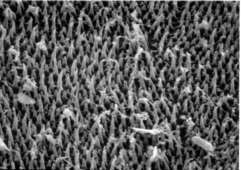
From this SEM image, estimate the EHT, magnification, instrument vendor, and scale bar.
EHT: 20 kV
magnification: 10 K X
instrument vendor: JEOL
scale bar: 1000 nm